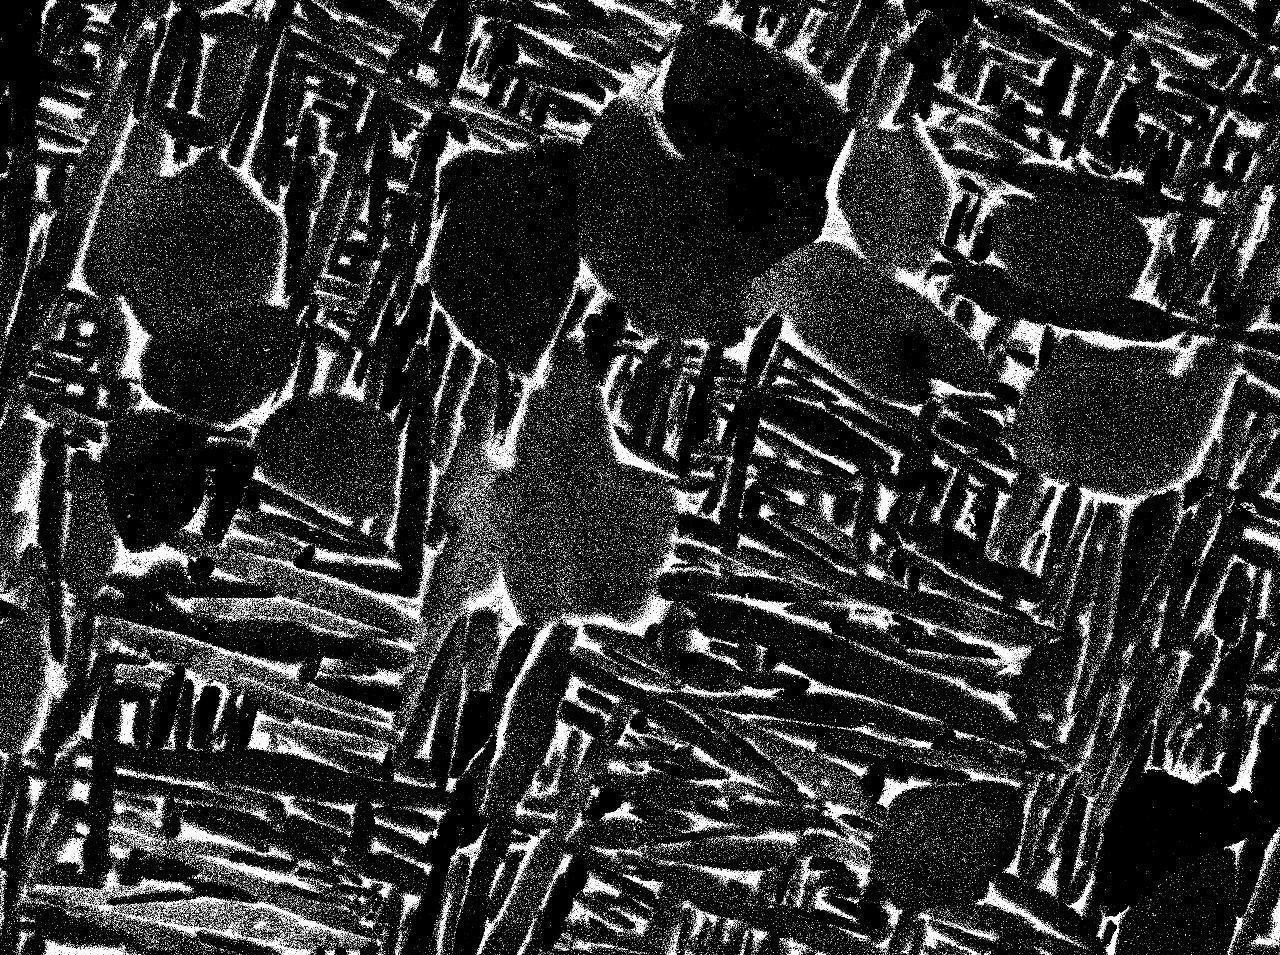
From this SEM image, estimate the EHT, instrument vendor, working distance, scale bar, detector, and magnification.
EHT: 15 kV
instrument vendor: JEOL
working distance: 10.1 mm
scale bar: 1000 nm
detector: COMPO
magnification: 3 K X